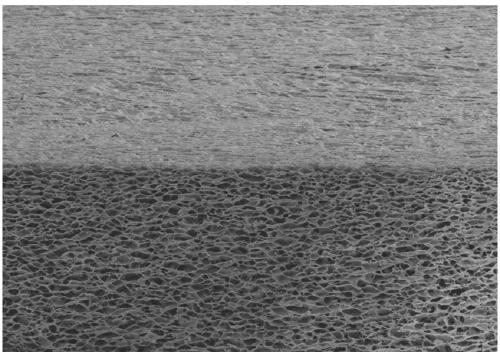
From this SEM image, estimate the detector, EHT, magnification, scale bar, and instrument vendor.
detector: SE(M)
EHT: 4 kV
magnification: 0.2 K X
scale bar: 200000 nm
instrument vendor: Hitachi